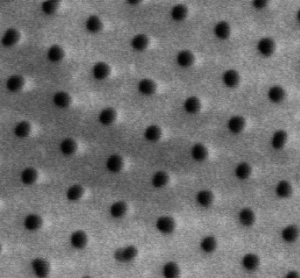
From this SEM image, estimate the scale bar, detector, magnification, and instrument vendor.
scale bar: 300 nm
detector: SE(M)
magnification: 150 K X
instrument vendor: Hitachi